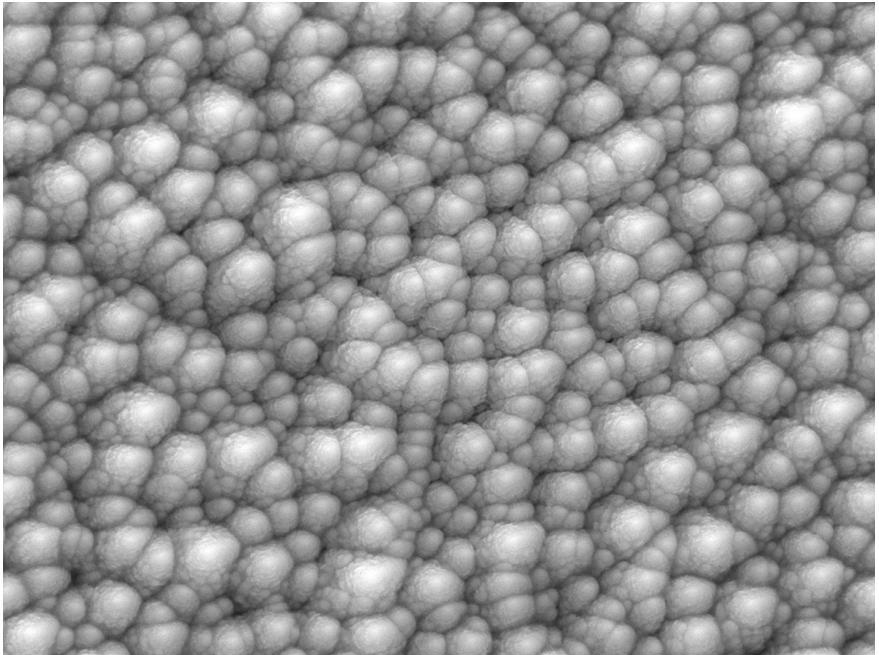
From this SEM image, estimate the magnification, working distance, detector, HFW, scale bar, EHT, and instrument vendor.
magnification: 50 K X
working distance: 15.39 mm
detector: SE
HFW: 5.54 µm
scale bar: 1000 nm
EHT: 20 kV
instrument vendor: Tescan